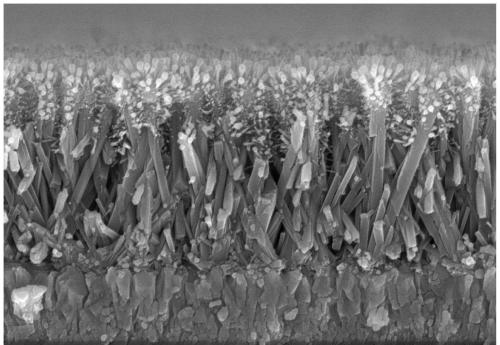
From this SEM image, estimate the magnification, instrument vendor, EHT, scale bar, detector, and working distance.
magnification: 25 K X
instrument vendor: Hitachi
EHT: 10 kV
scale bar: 2000 nm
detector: SE(M)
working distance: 10.1 mm